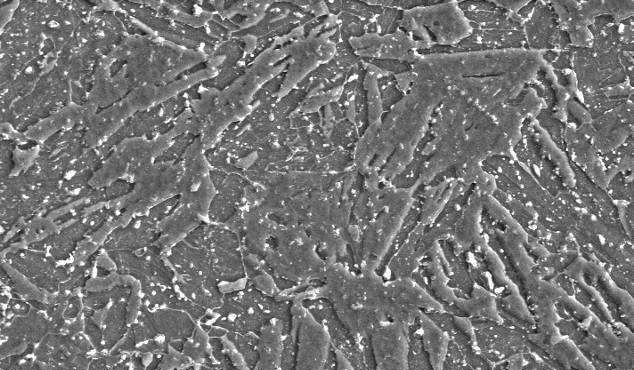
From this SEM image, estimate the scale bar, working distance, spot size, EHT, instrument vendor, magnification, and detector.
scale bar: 10000 nm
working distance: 11.2 mm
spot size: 4.2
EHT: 20 kV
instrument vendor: FEI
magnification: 1.5 K X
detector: SE